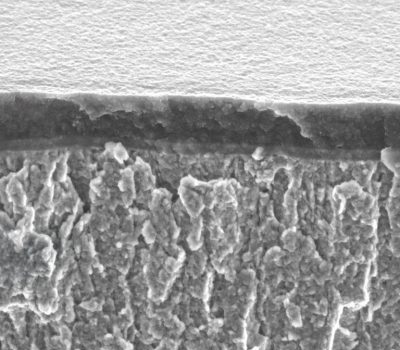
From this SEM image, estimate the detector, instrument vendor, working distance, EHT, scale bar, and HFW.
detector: SE2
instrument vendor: Zeiss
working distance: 3 mm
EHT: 5 kV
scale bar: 1000 nm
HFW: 11.43 µm